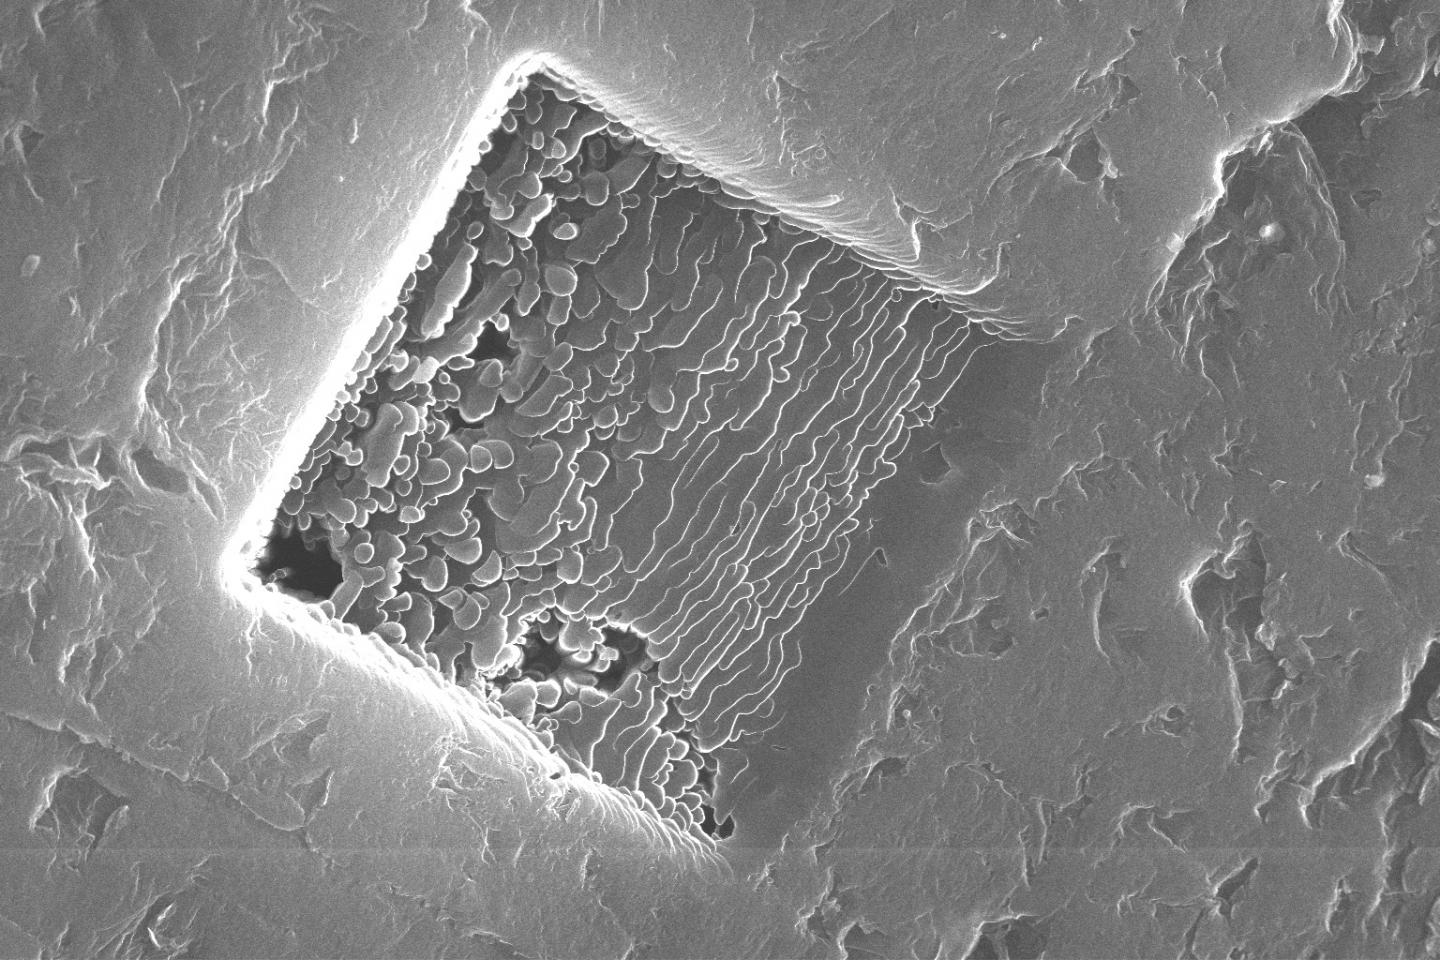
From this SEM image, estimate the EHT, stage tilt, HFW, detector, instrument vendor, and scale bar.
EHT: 30 kV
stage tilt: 0°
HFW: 34.5 µm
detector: SE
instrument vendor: FEI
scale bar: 10000 nm